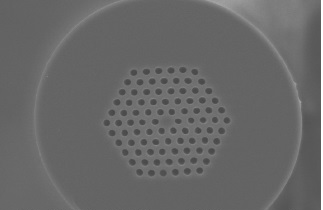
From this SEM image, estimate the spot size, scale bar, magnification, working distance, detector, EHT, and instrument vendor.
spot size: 4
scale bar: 20000 nm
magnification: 0.8 K X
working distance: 14.6 mm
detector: SE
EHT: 20 kV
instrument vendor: FEI